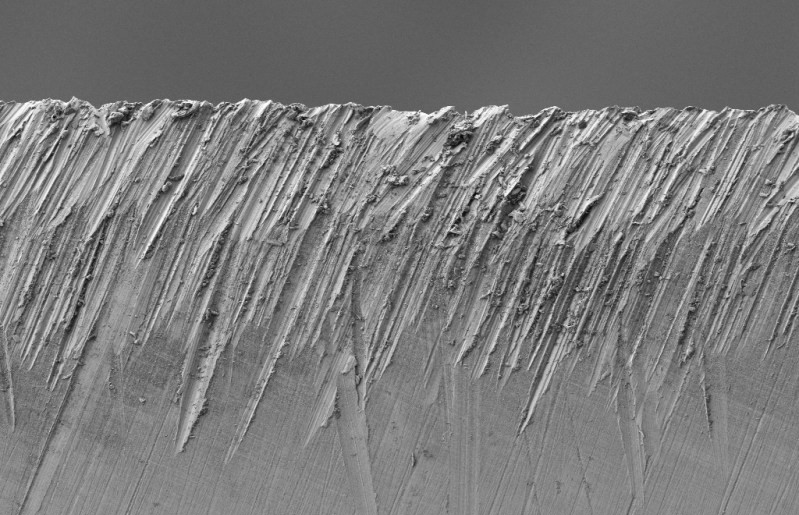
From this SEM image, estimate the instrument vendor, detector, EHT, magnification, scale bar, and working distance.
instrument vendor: Zeiss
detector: SE2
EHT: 1 kV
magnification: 1 K X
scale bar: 10000 nm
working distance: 3.8 mm